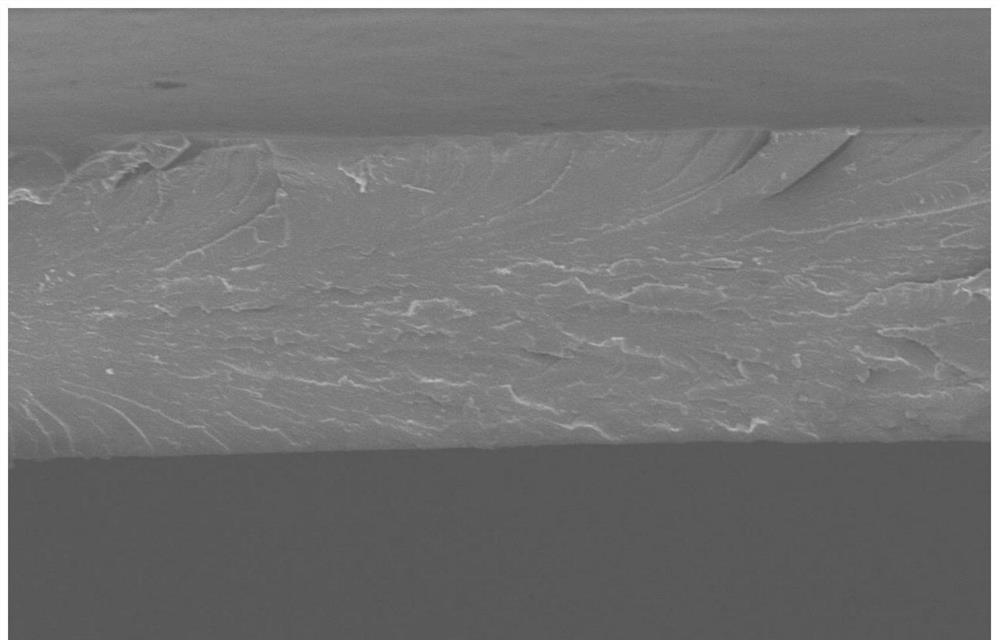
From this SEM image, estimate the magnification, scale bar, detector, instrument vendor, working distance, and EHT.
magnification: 0.6 K X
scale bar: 50000 nm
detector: SE(M)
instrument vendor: Hitachi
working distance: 10.8 mm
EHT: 5 kV